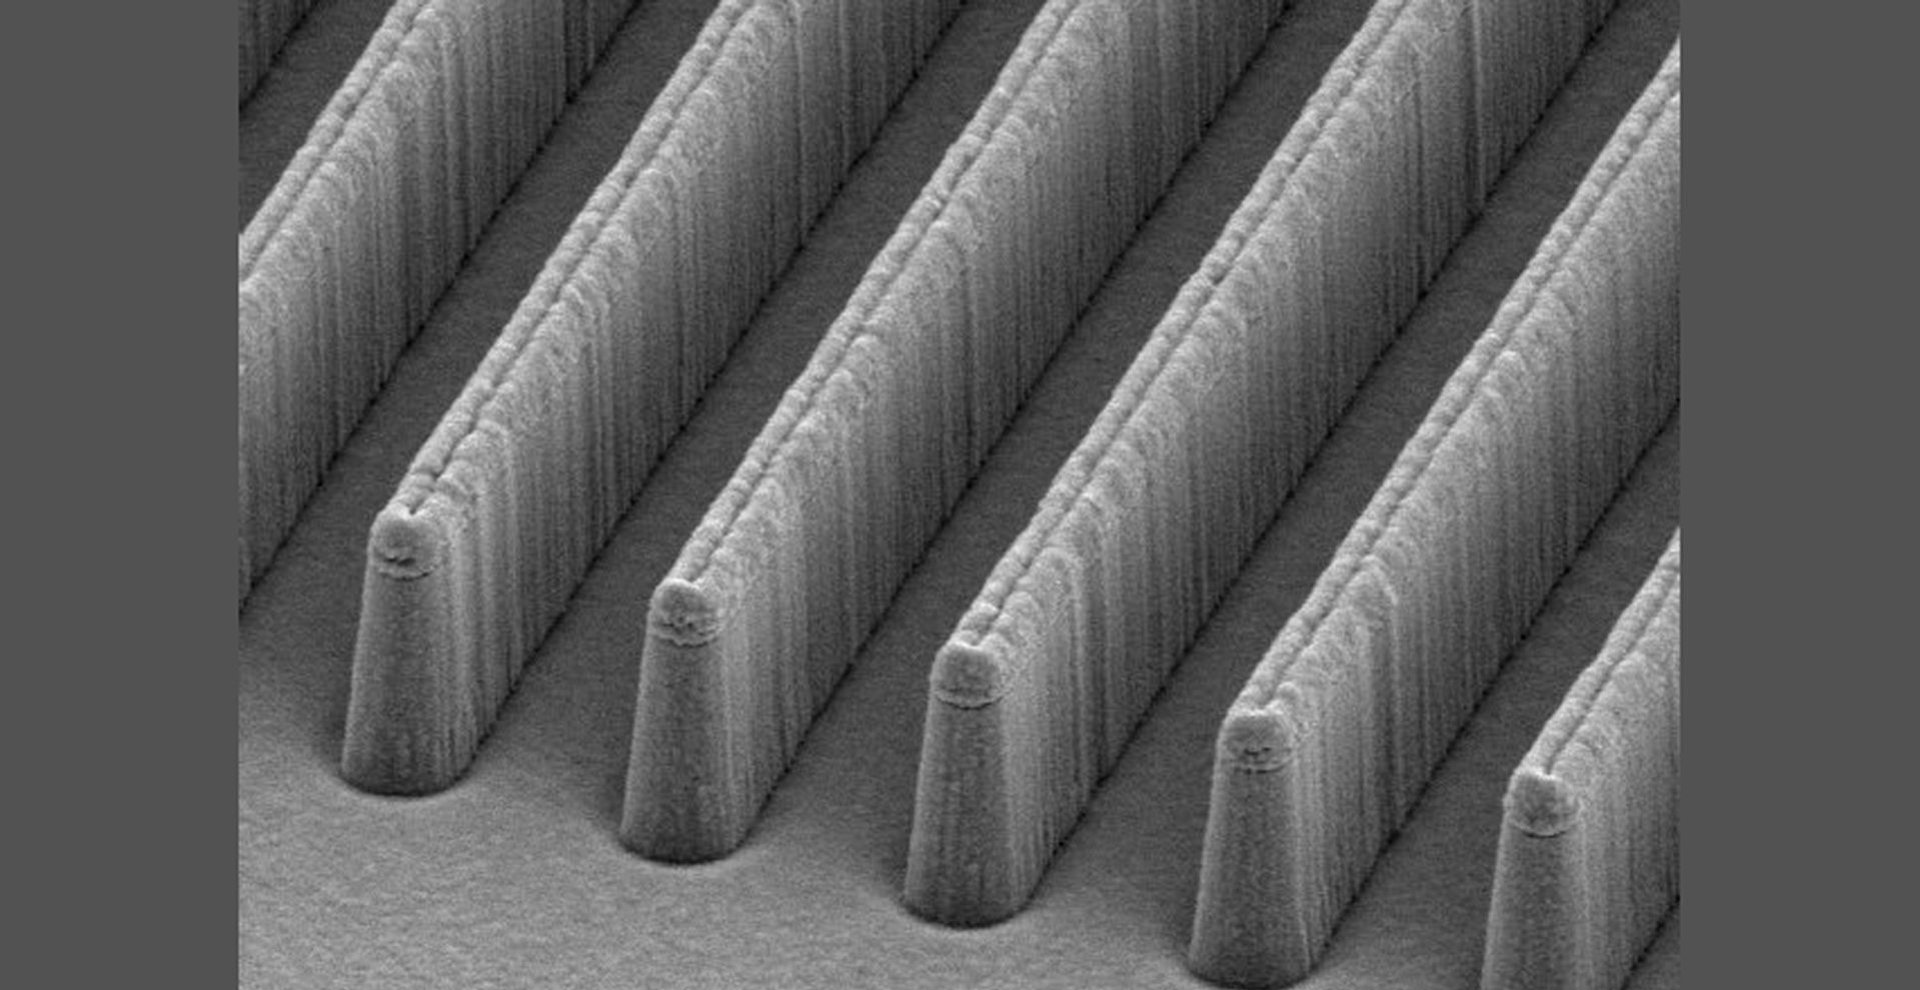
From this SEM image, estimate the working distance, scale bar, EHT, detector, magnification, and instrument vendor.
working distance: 39.8 mm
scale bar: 1500 nm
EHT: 5 kV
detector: SE(M)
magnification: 20 K X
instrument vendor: Hitachi